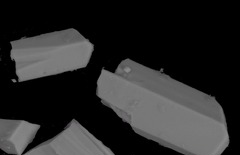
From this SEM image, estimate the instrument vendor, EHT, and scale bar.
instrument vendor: JEOL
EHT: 25 kV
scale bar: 20000 nm